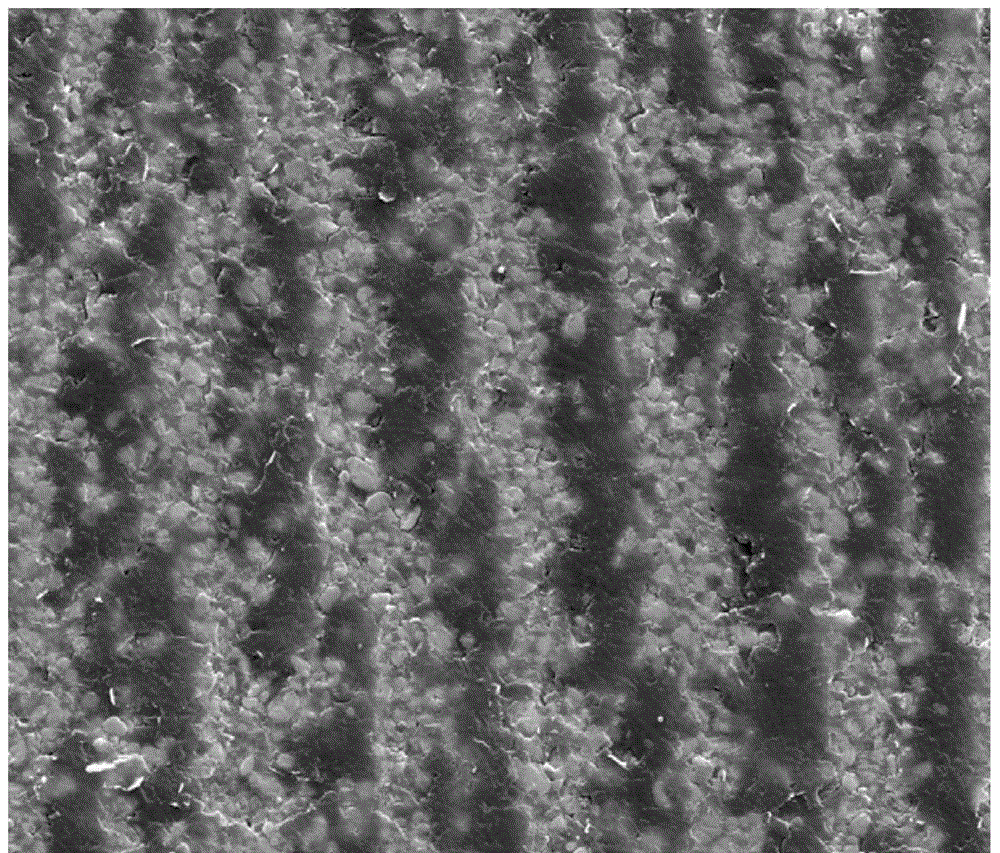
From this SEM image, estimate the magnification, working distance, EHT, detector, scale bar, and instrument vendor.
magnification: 4 K X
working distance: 10.5 mm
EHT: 30 kV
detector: SE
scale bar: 30000 nm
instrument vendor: FEI